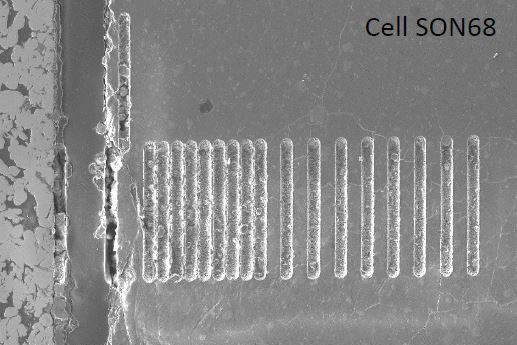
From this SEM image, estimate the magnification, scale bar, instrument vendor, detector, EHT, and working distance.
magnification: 0.04 K X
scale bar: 100000 nm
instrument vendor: JEOL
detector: LensSE LM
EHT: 15 kV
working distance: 12.2 mm